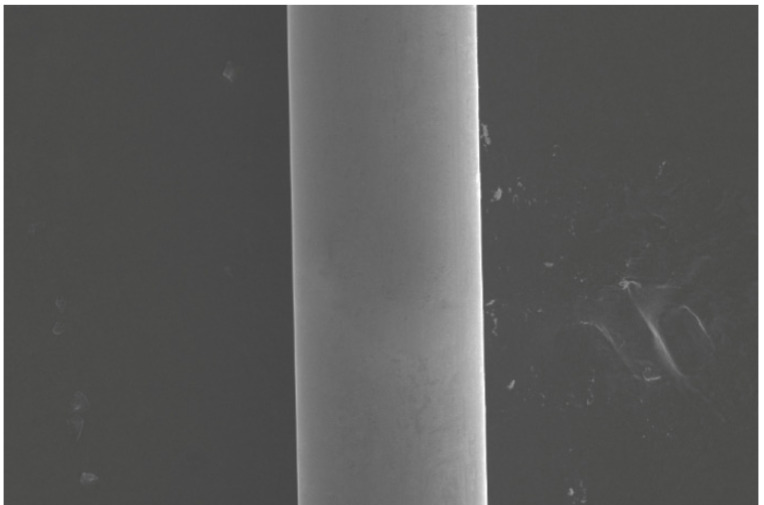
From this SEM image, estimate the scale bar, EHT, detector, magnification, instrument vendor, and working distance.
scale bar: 10000 nm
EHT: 15 kV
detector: SE2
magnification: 1.5 K X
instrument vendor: Zeiss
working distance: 2 mm